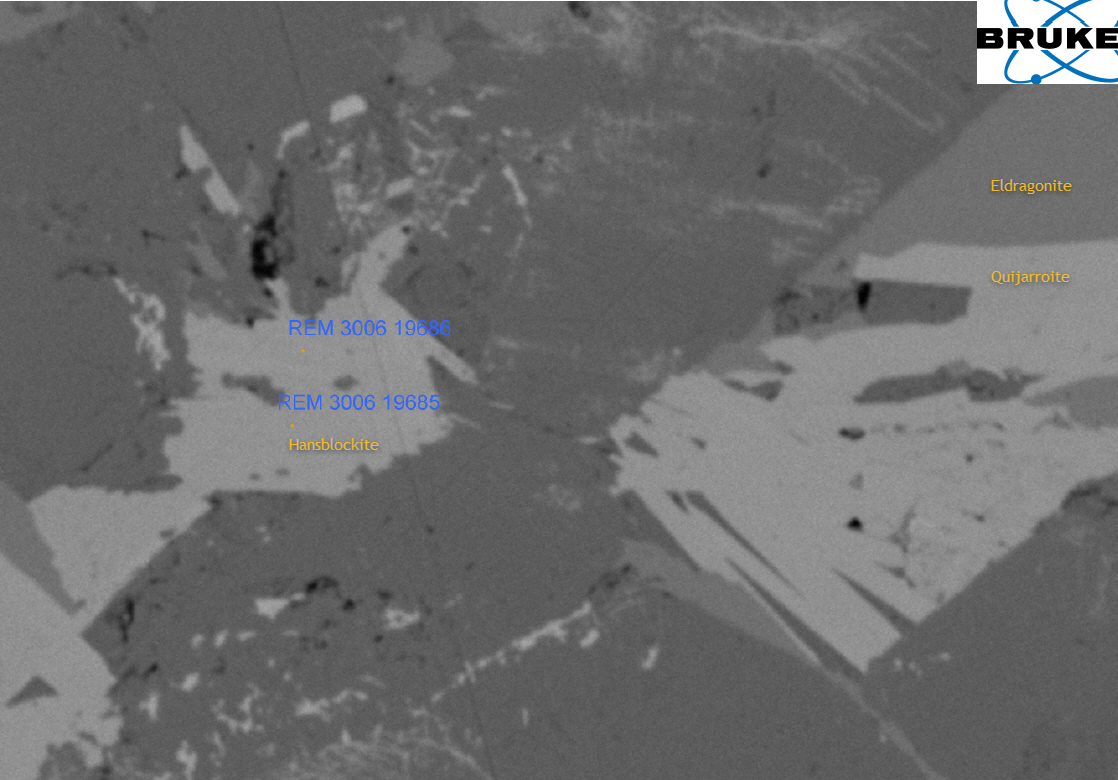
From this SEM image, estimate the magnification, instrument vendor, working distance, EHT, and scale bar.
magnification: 0.777 K X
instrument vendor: other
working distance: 11.2 mm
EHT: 15 kV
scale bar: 30000 nm